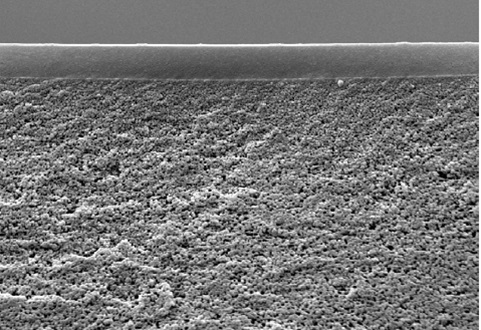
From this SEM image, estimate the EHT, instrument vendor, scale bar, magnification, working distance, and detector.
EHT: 20 kV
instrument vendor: Zeiss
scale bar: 2000 nm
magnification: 3 K X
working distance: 10 mm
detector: SE1